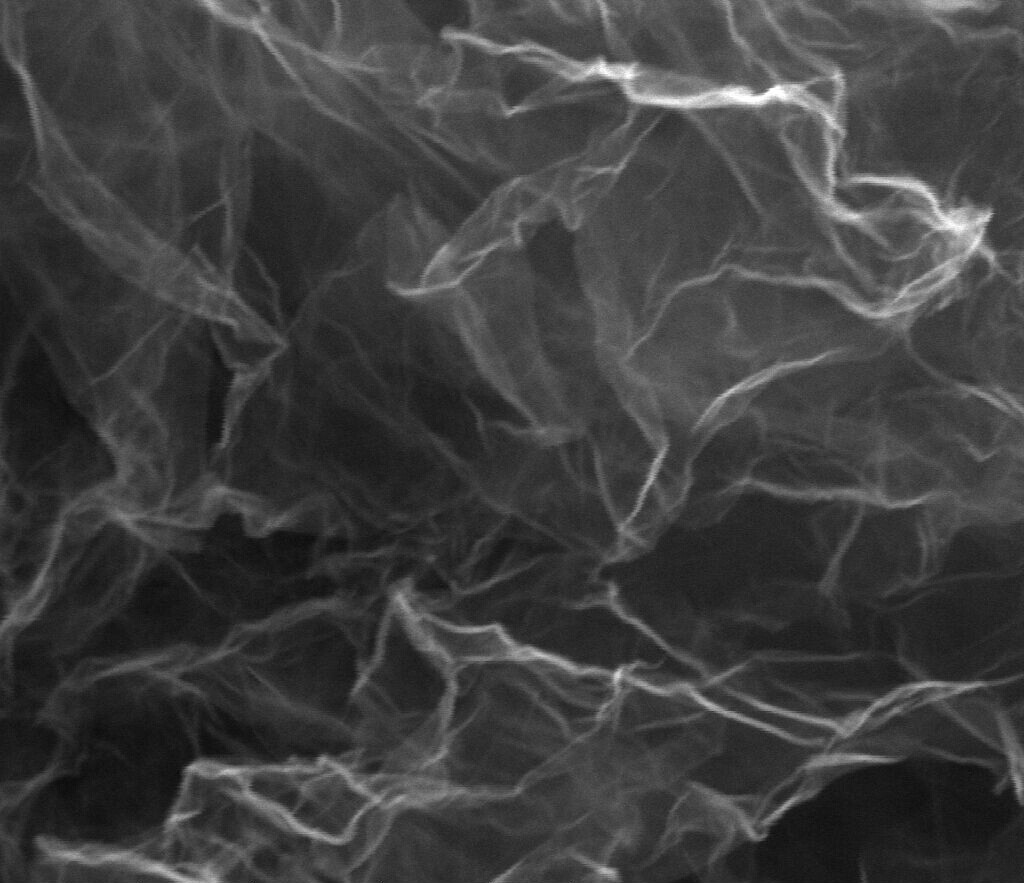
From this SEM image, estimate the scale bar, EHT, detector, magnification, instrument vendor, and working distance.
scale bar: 1000 nm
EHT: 20 kV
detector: SE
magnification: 100 K X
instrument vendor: FEI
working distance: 14.1 mm